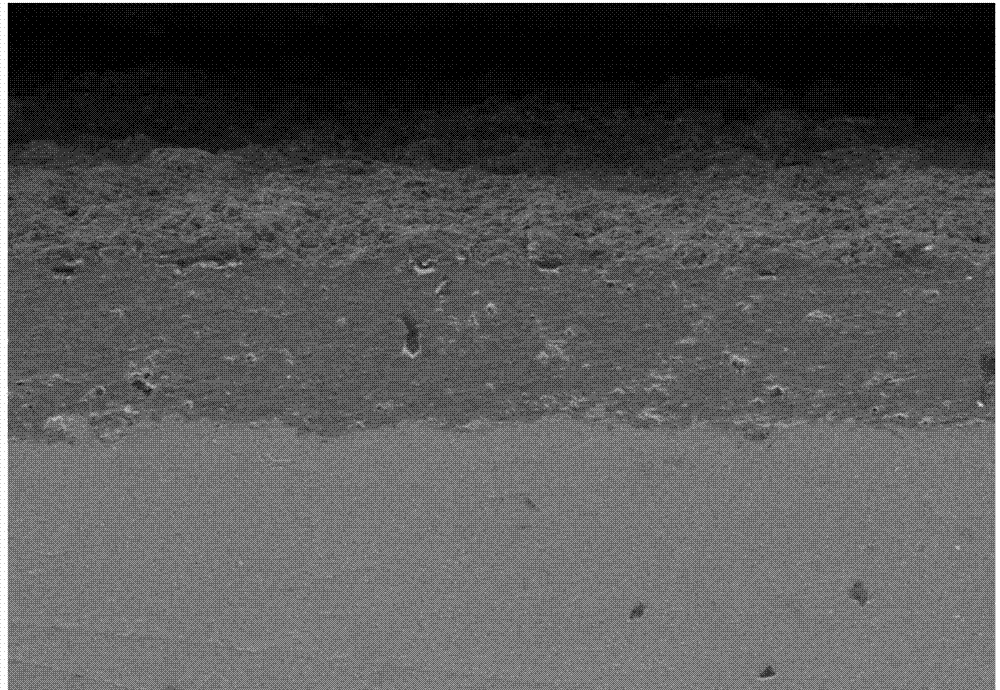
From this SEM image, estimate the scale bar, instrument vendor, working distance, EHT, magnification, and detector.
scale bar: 500000 nm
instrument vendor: Hitachi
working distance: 11 mm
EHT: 15 kV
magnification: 0.1 K X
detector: SE(M)